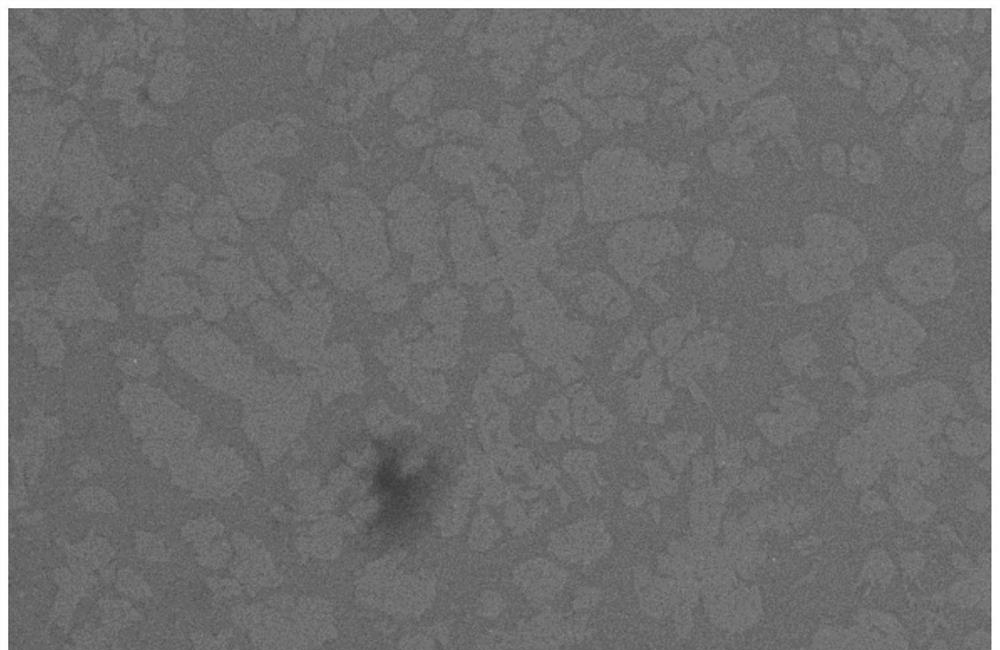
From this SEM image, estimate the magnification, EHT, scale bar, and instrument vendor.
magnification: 0.5 K X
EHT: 20 kV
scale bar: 50000 nm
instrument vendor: JEOL